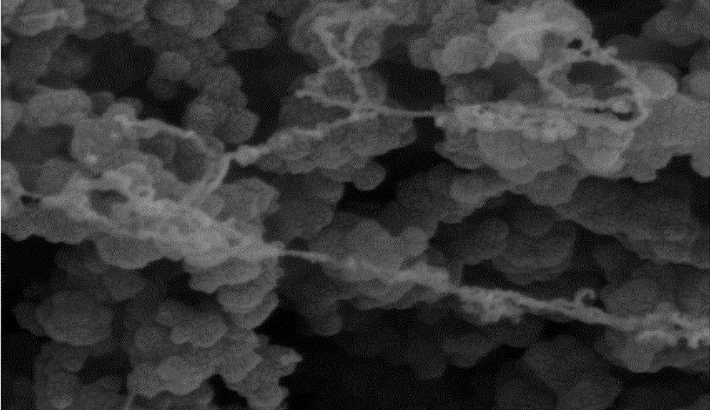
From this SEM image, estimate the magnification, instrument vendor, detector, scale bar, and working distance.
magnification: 100 K X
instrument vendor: Tescan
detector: SE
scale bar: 500 nm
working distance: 11.42 mm